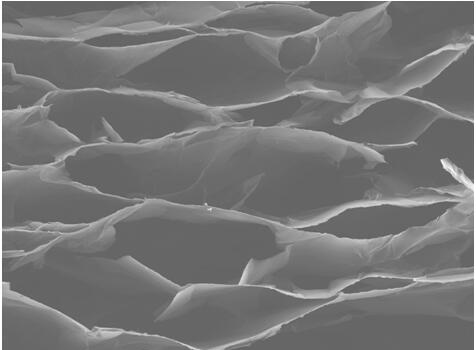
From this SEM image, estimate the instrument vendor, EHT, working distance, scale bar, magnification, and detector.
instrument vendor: JEOL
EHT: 15 kV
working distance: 8 mm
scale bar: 100000 nm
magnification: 0.06 K X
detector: SEI